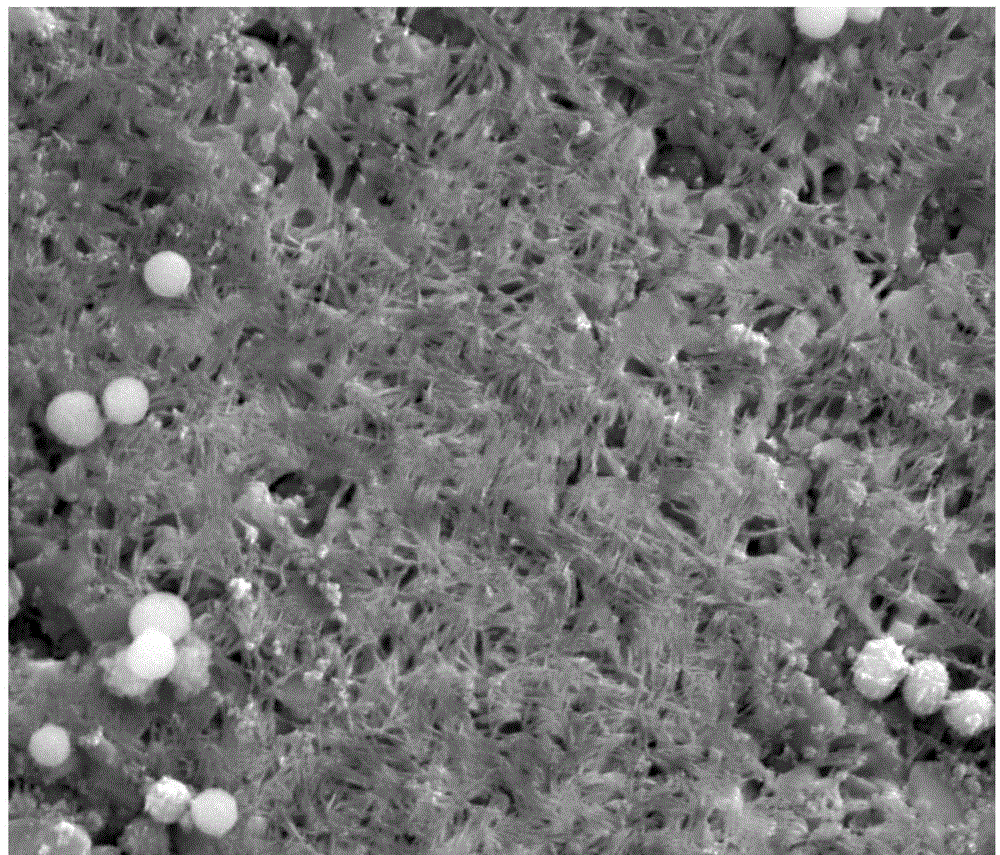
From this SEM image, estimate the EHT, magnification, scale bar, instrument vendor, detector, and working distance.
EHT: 10 kV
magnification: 30 K X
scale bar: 3000 nm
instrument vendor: FEI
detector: ETD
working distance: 6.3 mm